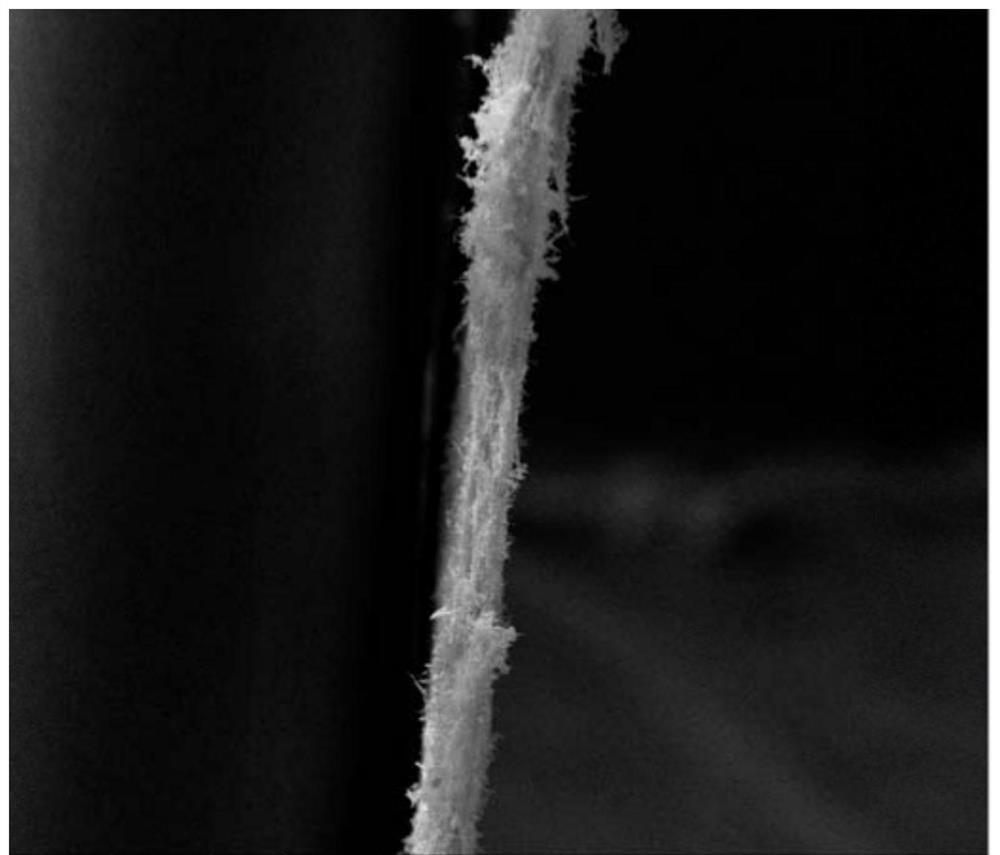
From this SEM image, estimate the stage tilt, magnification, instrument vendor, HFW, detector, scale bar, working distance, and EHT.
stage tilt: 0°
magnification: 0.4 K X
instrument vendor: FEI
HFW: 746 µm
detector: ETD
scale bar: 300000 nm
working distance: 4.6 mm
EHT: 20 kV